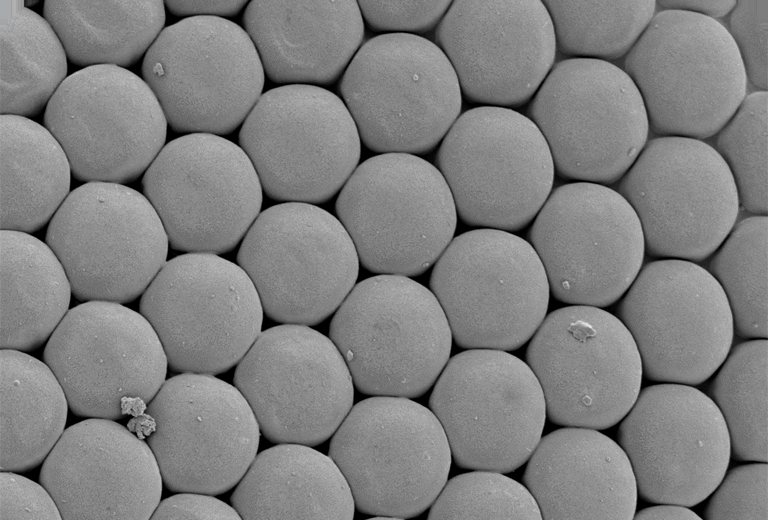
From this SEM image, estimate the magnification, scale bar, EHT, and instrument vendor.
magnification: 1 K X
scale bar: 10000 nm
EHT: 5 kV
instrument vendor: JEOL